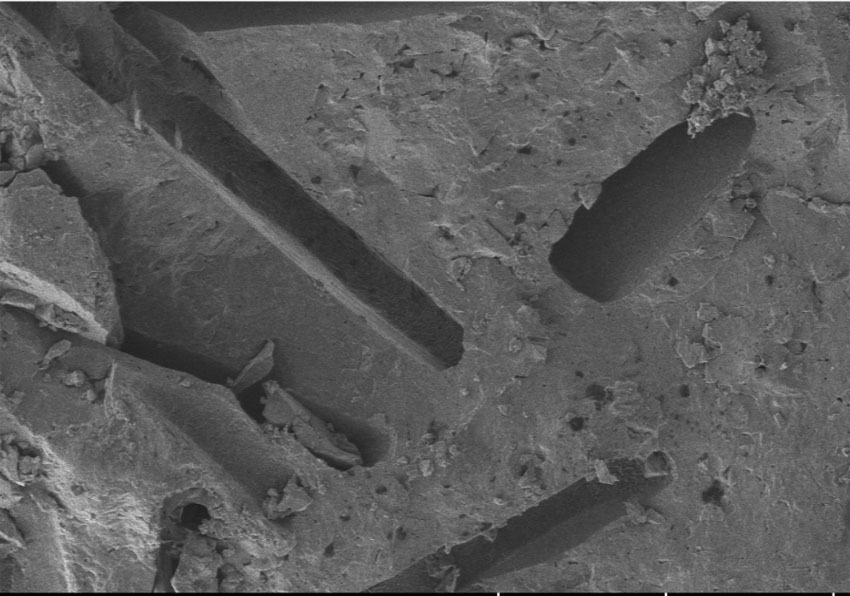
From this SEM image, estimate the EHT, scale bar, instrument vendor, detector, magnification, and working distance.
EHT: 5 kV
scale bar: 200000 nm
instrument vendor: Hitachi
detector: LM(L)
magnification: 0.25 K X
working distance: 15.5 mm